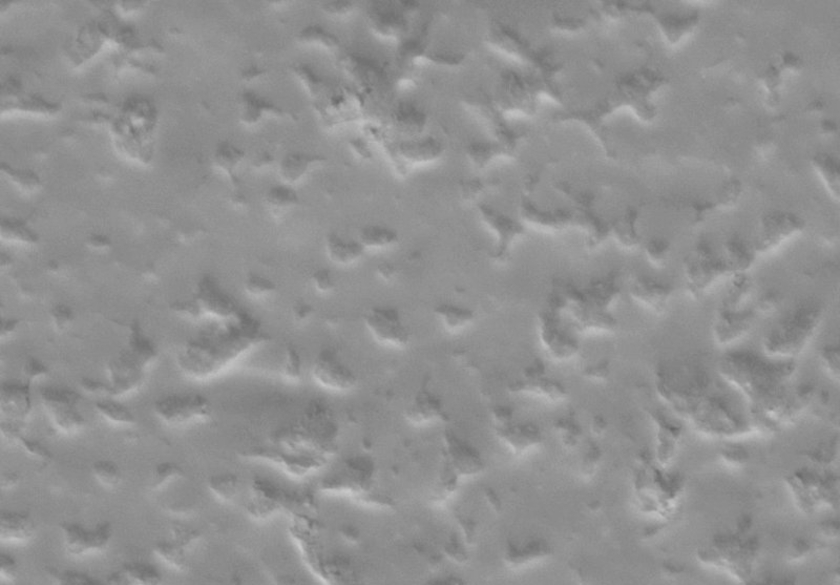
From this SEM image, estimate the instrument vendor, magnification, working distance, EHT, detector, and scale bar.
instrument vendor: Zeiss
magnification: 10 K X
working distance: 12.2 mm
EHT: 5 kV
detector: SE2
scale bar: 1000 nm